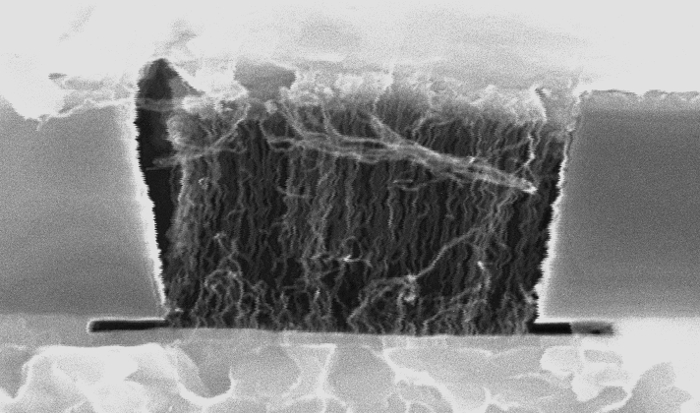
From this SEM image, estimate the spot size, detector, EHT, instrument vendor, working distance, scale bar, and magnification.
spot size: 2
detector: SE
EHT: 15 kV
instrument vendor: FEI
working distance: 11 mm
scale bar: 500 nm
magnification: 80 K X